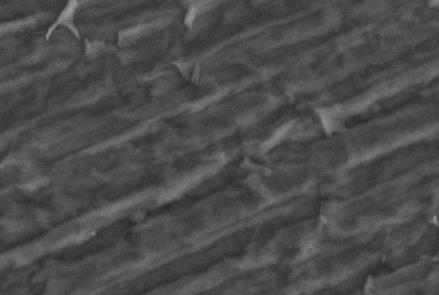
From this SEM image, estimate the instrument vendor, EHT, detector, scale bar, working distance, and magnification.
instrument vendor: JEOL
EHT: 15 kV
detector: BSE1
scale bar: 20000 nm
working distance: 15.7 mm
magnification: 2.5 K X